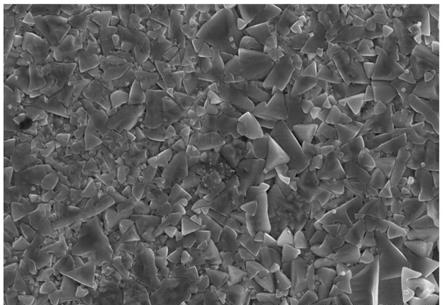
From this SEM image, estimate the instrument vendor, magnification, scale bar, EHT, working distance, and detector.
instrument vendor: Hitachi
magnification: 4.5 K X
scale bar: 10000 nm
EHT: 15 kV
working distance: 9.3 mm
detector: SE(UL)